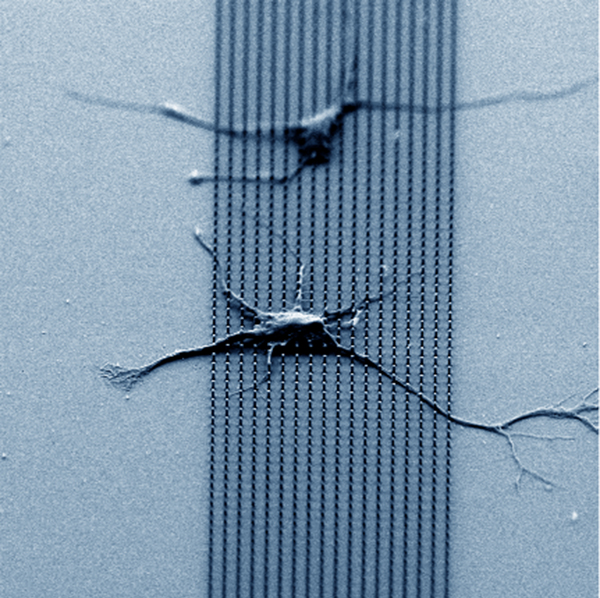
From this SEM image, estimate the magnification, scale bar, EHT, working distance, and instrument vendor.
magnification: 1 K X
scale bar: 20000 nm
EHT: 5 kV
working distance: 3 mm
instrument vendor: Hitachi